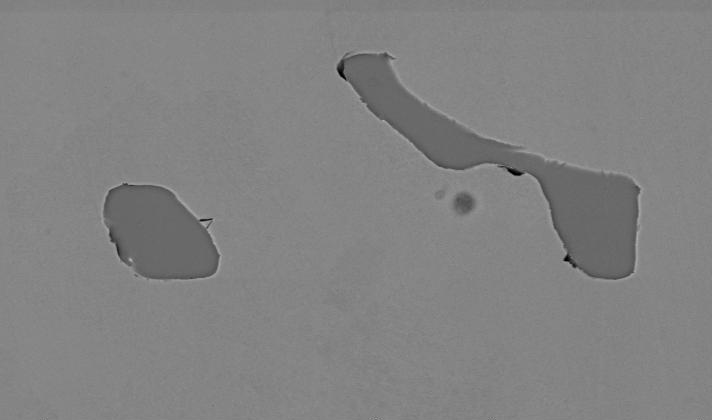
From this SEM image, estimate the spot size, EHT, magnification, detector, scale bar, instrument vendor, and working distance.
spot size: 4.8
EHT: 20 kV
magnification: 0.75 K X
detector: BSE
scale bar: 20000 nm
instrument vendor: FEI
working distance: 10.9 mm